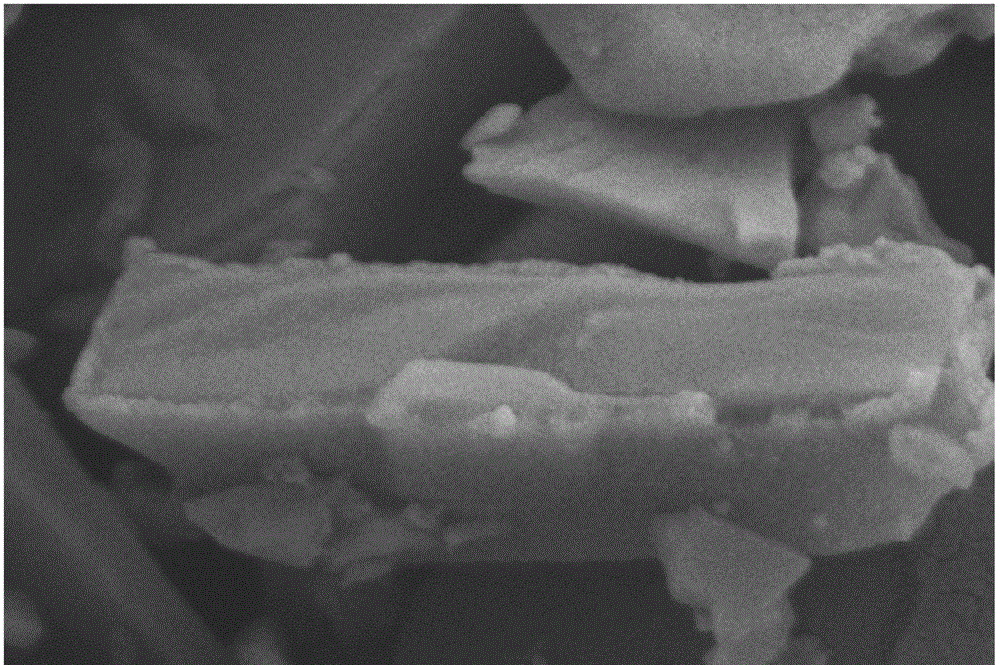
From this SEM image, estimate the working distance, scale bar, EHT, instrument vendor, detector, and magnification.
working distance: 3.4 mm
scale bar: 200 nm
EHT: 5 kV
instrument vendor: Zeiss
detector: SE2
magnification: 50 K X